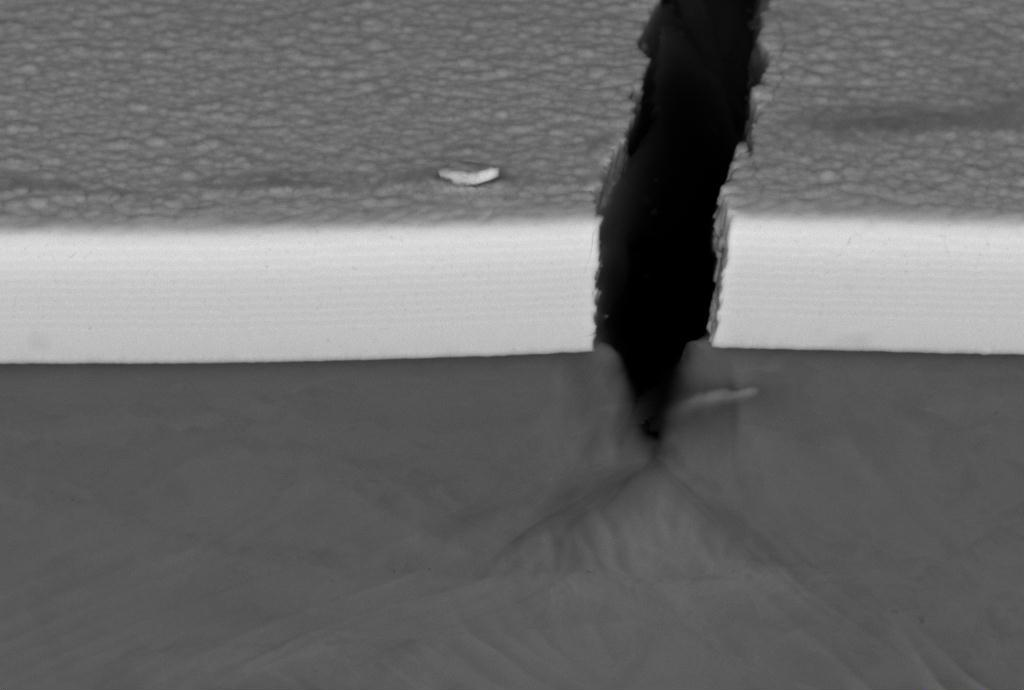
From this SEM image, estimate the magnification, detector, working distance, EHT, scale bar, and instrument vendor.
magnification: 10 K X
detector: CZ BSD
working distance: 7.5 mm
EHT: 20 kV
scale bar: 1000 nm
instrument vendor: Zeiss